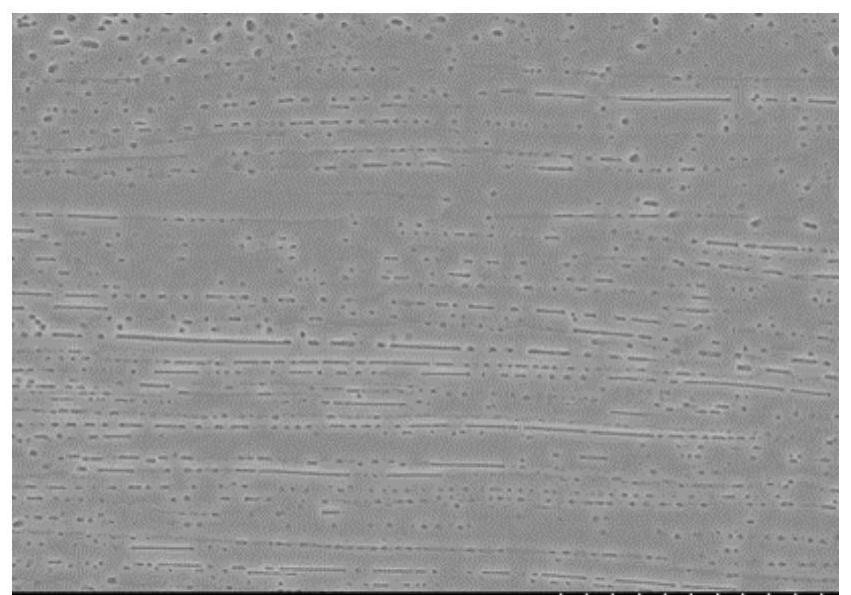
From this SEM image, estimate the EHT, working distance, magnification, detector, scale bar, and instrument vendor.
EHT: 15 kV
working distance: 17.5 mm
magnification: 2 K X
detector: SE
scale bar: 20000 nm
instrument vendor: Hitachi